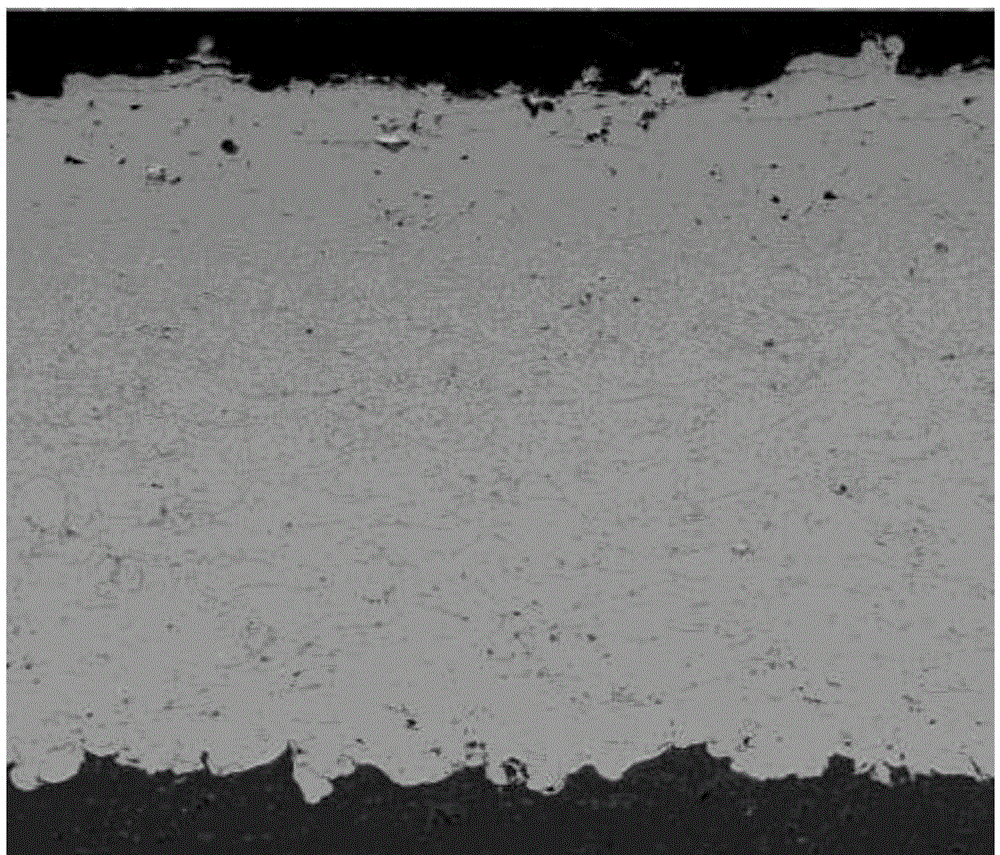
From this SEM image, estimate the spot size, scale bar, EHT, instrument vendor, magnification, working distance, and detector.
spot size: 4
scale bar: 100000 nm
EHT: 30 kV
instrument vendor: FEI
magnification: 0.4 K X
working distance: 11 mm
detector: BSE